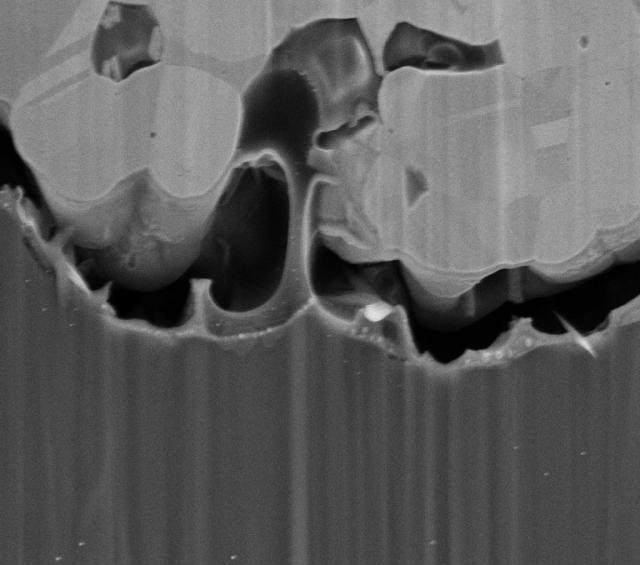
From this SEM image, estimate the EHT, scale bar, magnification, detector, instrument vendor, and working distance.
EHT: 4 kV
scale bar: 1000 nm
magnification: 13 K X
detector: SE2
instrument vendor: Zeiss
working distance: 5.4 mm